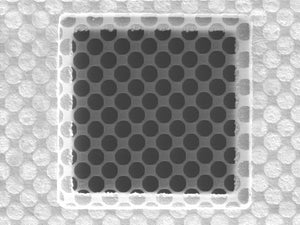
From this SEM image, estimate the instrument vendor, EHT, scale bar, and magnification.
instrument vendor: other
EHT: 5 kV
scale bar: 20000 nm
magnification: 1.65 K X